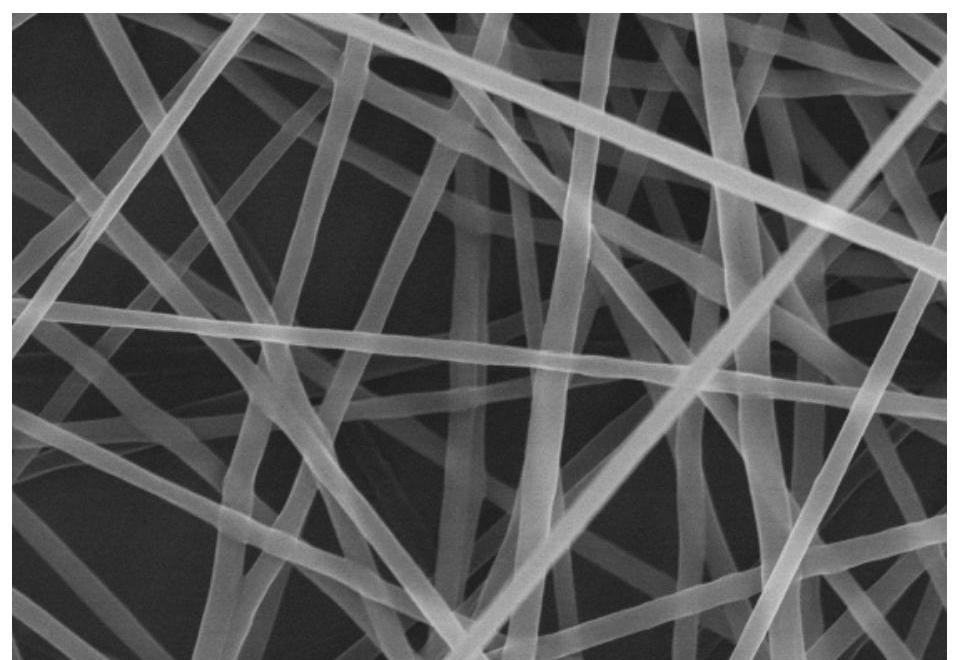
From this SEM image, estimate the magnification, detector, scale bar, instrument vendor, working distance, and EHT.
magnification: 10 K X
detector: SE(M)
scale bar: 5000 nm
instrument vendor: Hitachi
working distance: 6.2 mm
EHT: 15 kV